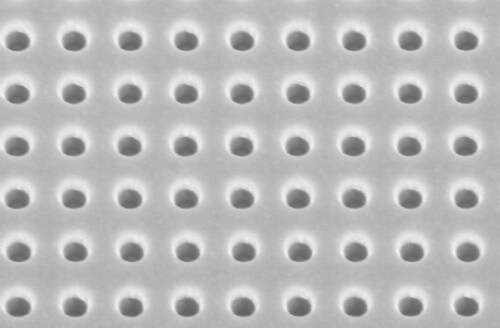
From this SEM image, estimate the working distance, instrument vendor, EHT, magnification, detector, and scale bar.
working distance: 10.5 mm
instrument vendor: Zeiss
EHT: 10 kV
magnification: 25.56 K X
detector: InLens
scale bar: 200 nm